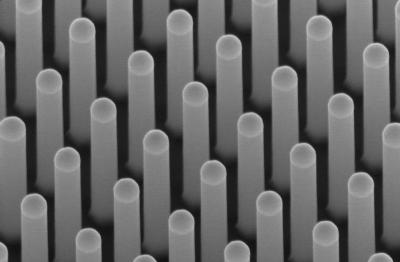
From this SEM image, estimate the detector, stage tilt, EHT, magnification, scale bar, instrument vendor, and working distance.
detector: InLens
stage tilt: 30°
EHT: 10 kV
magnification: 100.68 K X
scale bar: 1000 nm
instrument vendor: Zeiss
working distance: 6.7 mm